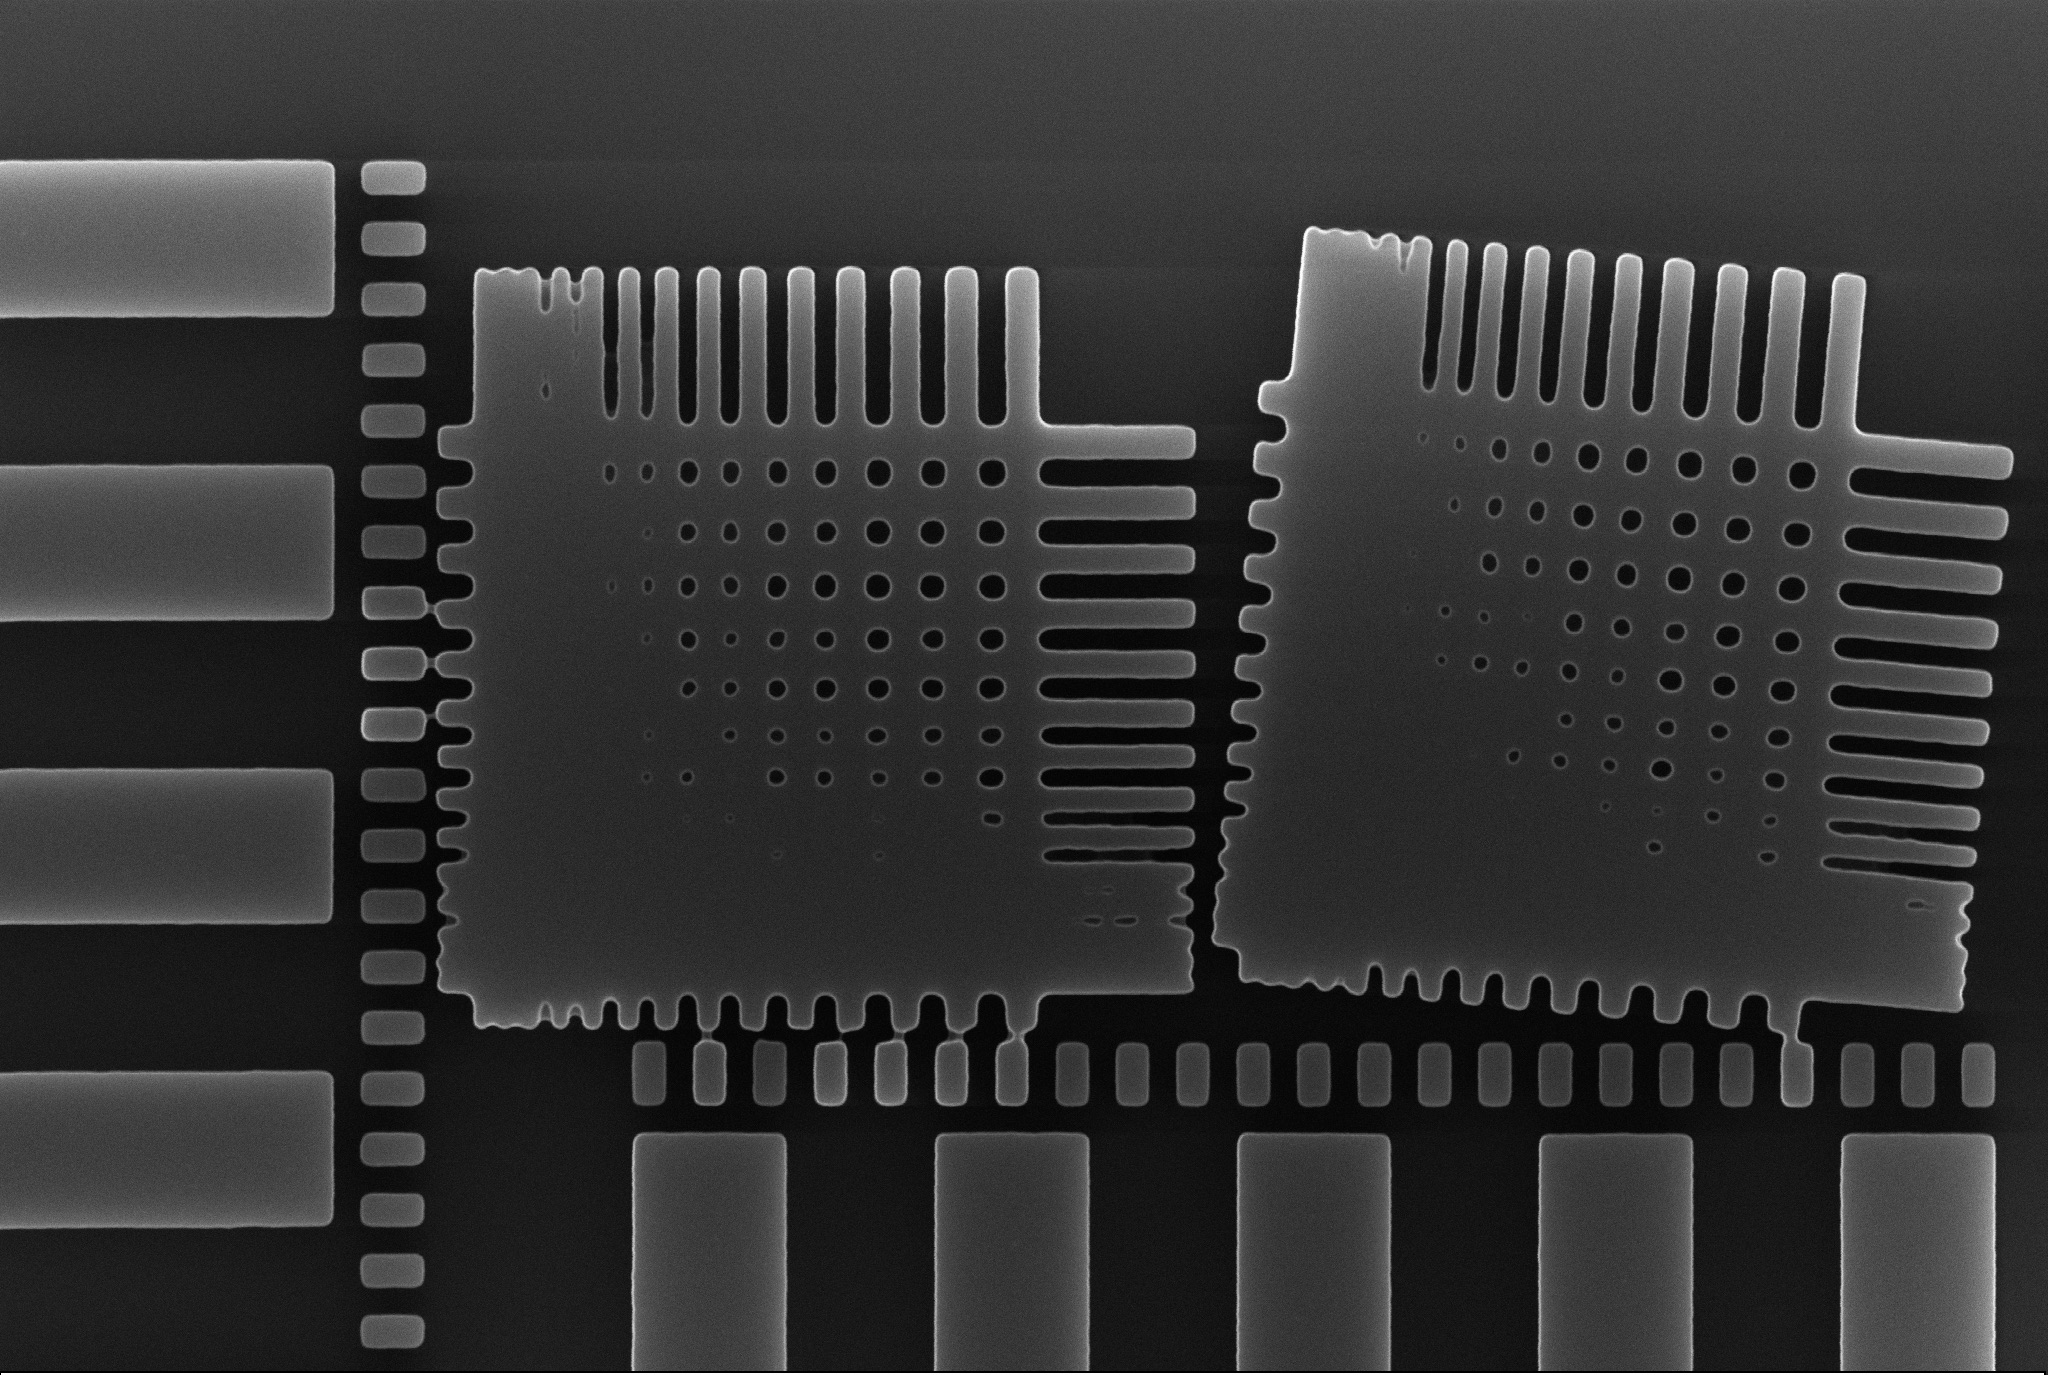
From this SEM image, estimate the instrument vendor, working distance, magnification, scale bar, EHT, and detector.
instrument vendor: Zeiss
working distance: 6.7 mm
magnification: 17.01 K X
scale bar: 1000 nm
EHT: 15 kV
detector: InLens